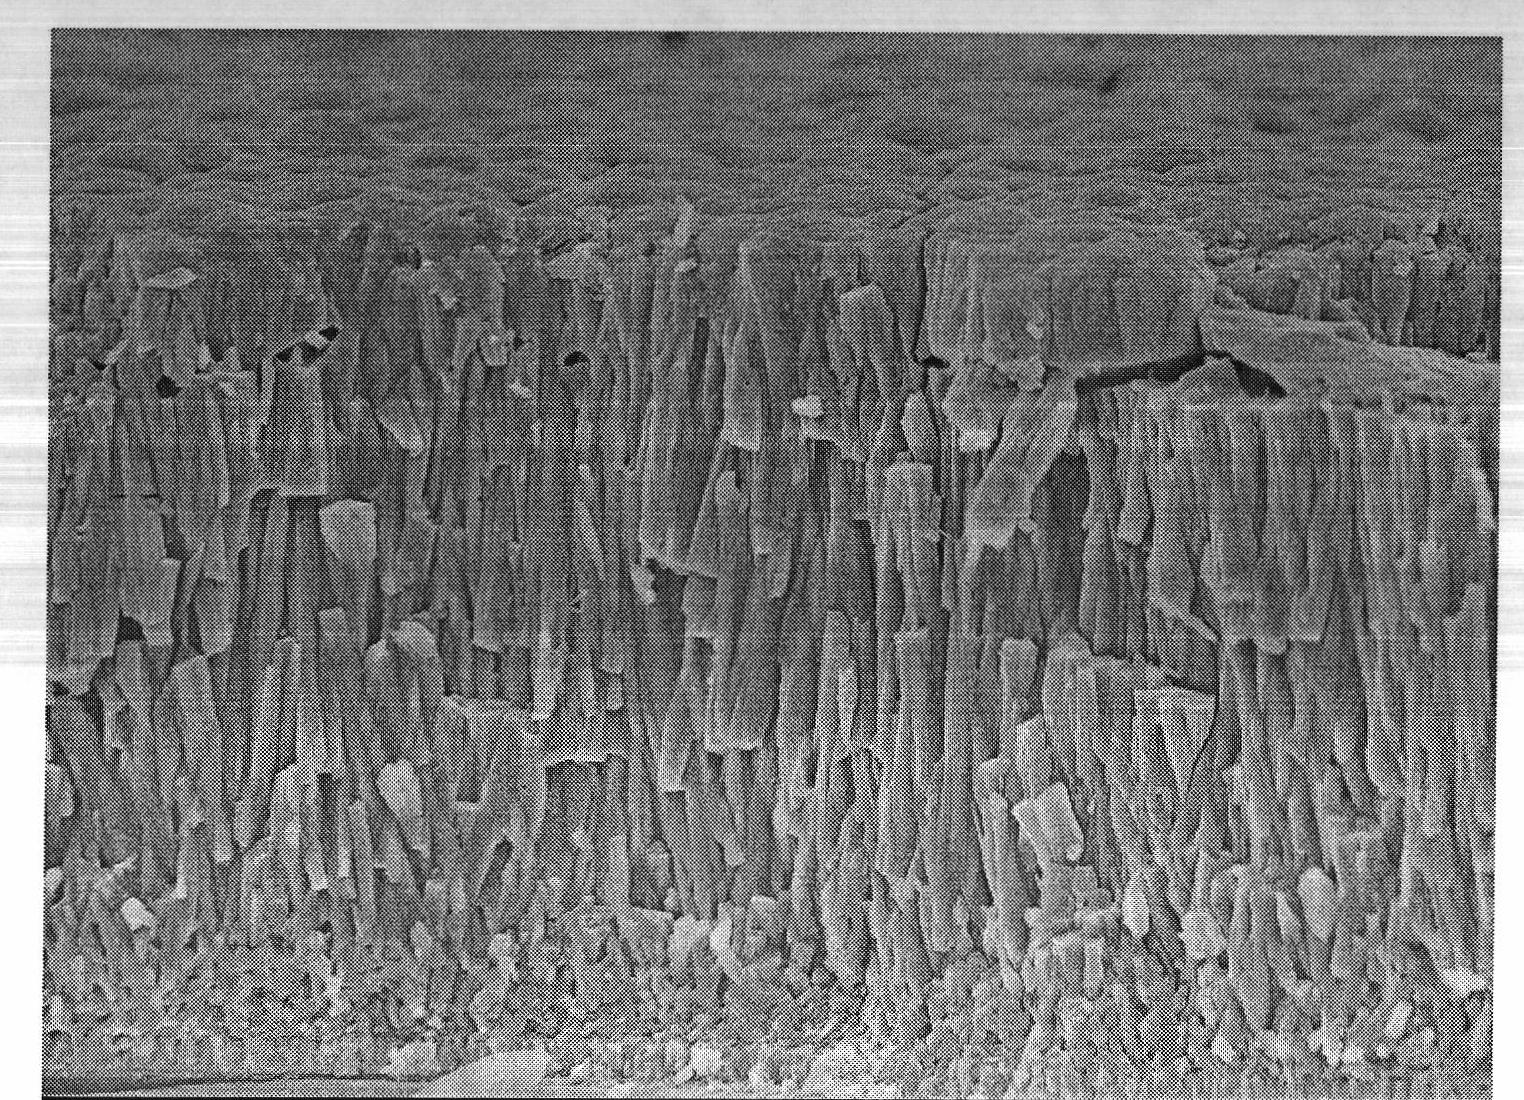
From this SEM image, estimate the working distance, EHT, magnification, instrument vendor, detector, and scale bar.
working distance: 10.3 mm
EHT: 5 kV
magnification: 6 K X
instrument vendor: JEOL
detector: SEI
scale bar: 1000 nm